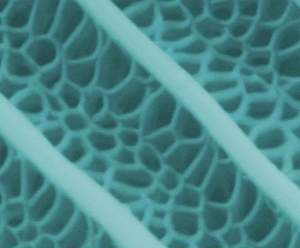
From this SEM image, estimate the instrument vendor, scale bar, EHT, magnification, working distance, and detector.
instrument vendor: FEI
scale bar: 2000 nm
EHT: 20 kV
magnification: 40 K X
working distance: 10.9 mm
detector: LFD